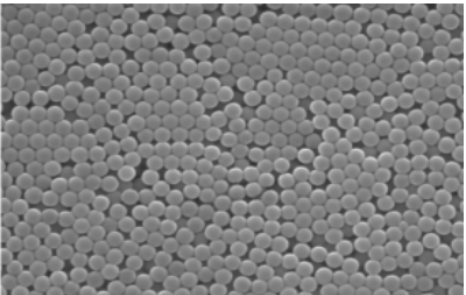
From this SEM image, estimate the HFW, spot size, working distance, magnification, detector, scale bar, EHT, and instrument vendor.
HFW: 16.6 µm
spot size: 4.5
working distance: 9.8 mm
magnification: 25 K X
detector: SE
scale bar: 5000 nm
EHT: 30 kV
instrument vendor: FEI